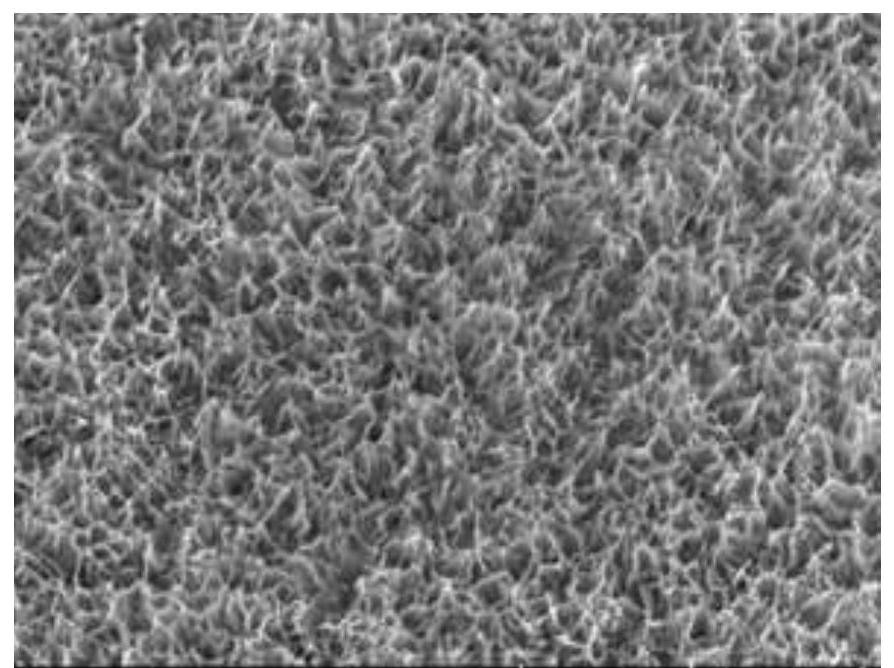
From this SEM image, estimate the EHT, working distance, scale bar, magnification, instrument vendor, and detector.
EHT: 5 kV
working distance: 8.7 mm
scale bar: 1000 nm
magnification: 50 K X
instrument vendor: Hitachi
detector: SE(UL)